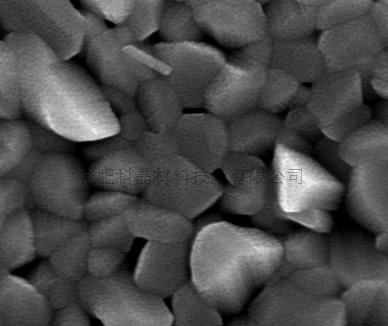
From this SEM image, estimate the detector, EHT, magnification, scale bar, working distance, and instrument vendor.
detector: TLD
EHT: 20 kV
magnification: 40 K X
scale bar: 1000 nm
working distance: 6 mm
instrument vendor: FEI